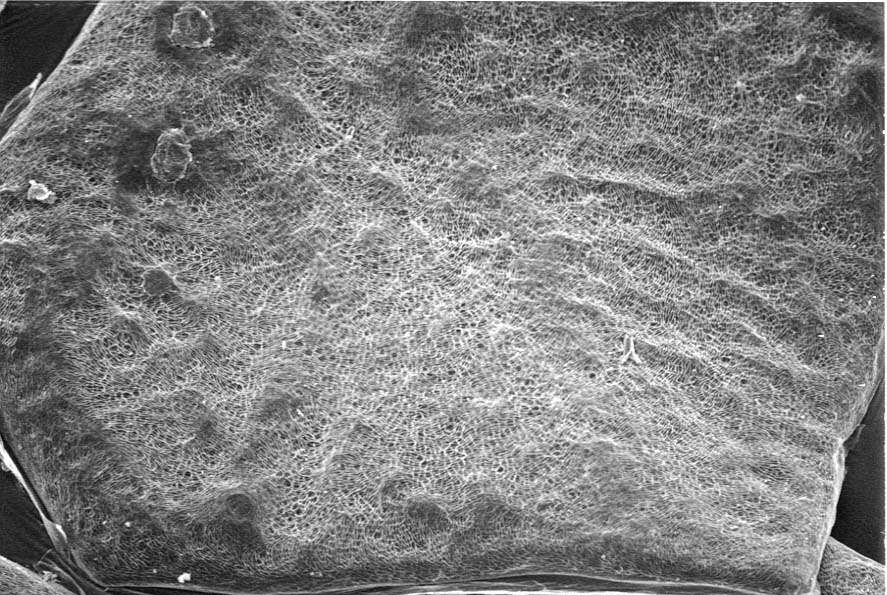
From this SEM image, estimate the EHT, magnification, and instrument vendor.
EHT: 20 kV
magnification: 0.052 K X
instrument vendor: JEOL